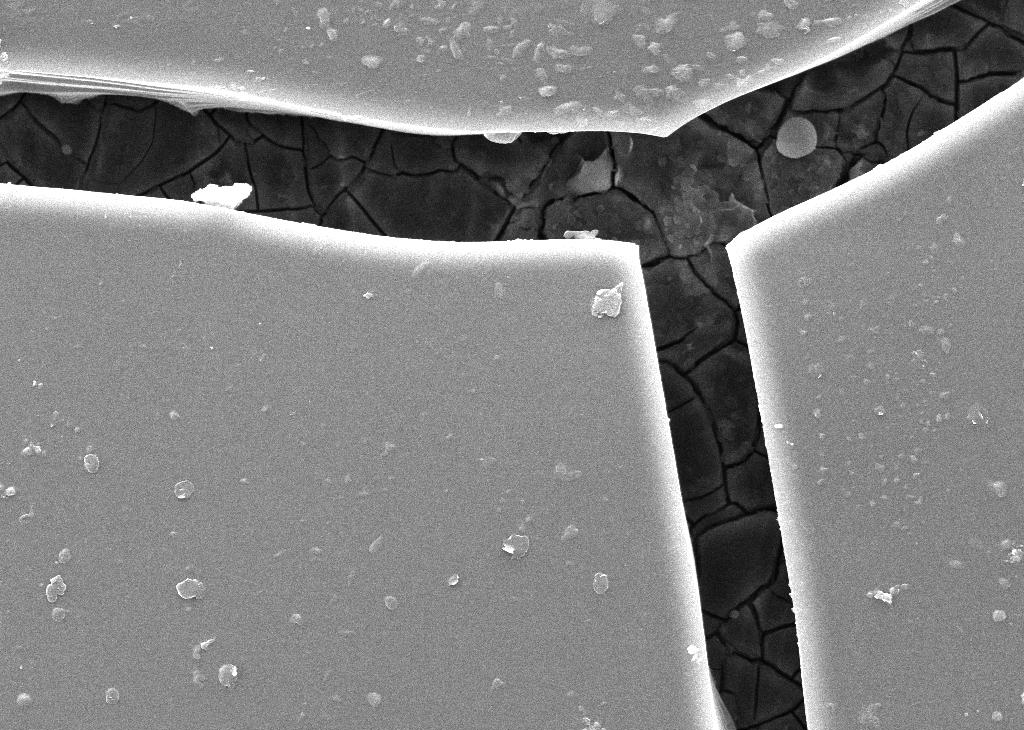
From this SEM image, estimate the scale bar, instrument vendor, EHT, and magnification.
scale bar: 20000 nm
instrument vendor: Tescan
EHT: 15 kV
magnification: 3 K X